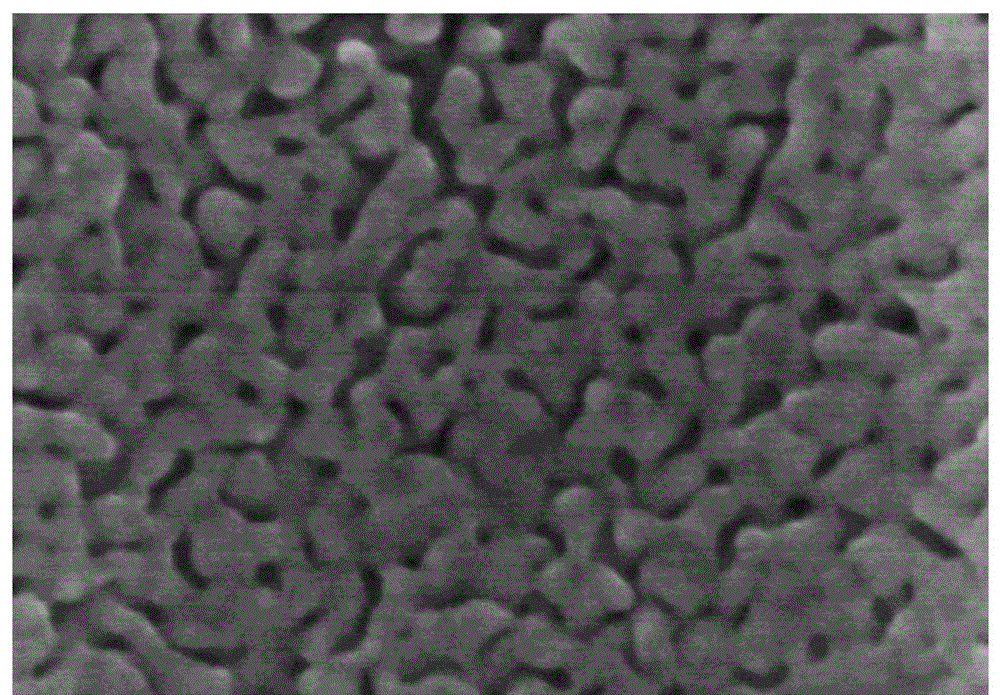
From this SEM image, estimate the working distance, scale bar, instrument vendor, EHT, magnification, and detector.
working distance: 9.2 mm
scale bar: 200 nm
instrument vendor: Hitachi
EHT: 15 kV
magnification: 200 K X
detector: SE(M)